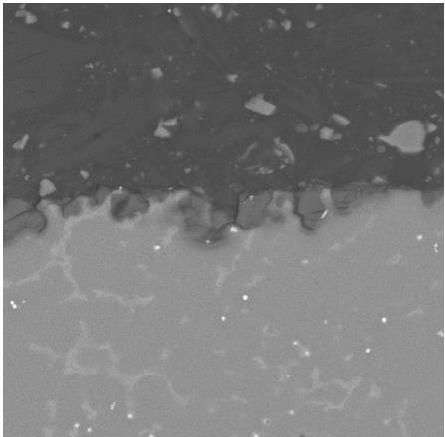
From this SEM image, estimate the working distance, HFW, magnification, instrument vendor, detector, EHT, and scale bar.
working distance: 18.03 mm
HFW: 207 µm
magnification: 1 K X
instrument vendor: Tescan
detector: BSE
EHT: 30 kV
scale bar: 50000 nm